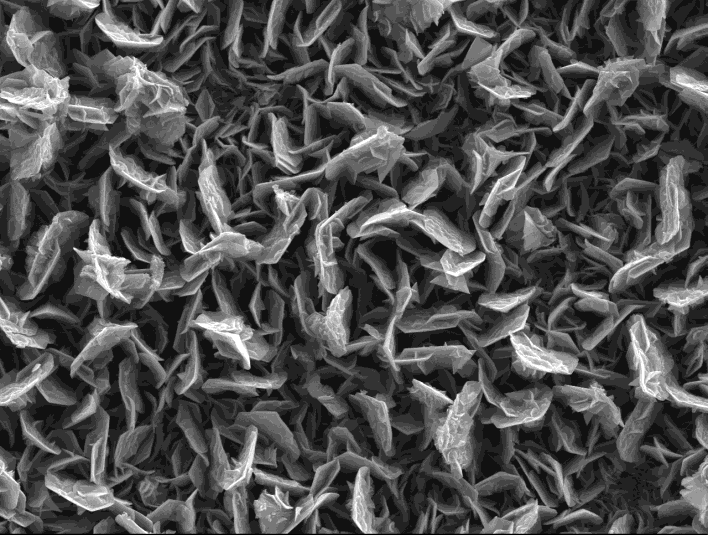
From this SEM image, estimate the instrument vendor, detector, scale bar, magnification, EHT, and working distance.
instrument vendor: JEOL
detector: SEI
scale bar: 1000 nm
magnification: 5 K X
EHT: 15 kV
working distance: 10.1 mm